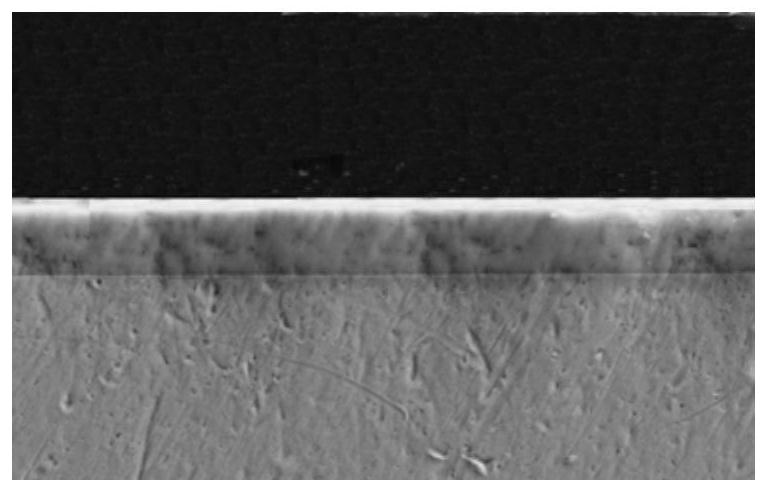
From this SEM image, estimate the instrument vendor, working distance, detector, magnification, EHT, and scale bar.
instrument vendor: Zeiss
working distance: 10.2 mm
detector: SE2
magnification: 1.47 K X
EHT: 20 kV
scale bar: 10000 nm